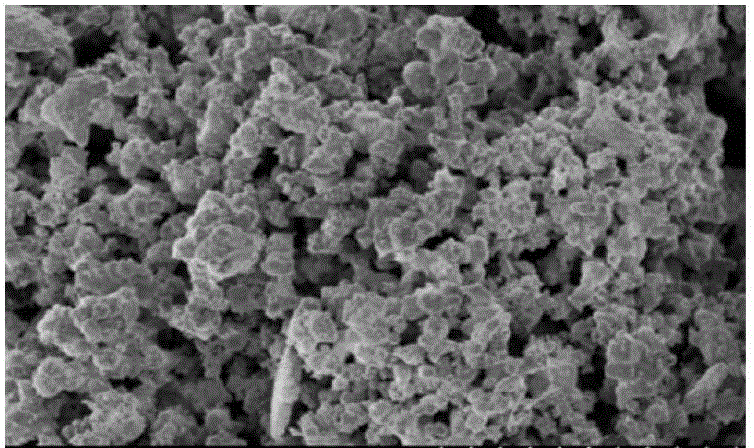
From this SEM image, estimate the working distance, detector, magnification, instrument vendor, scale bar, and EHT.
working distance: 6.9 mm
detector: SE(U)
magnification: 10 K X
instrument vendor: Hitachi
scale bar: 5000 nm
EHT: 1 kV